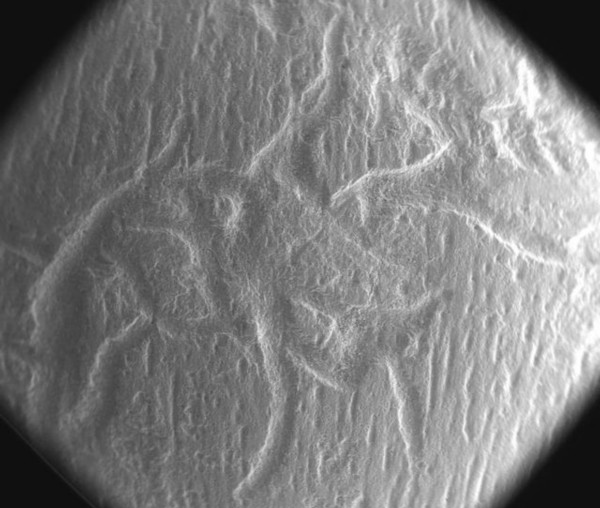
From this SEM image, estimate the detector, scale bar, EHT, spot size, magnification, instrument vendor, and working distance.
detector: LFD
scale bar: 2000000 nm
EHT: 30 kV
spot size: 4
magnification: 0.046 K X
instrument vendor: FEI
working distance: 10.3 mm